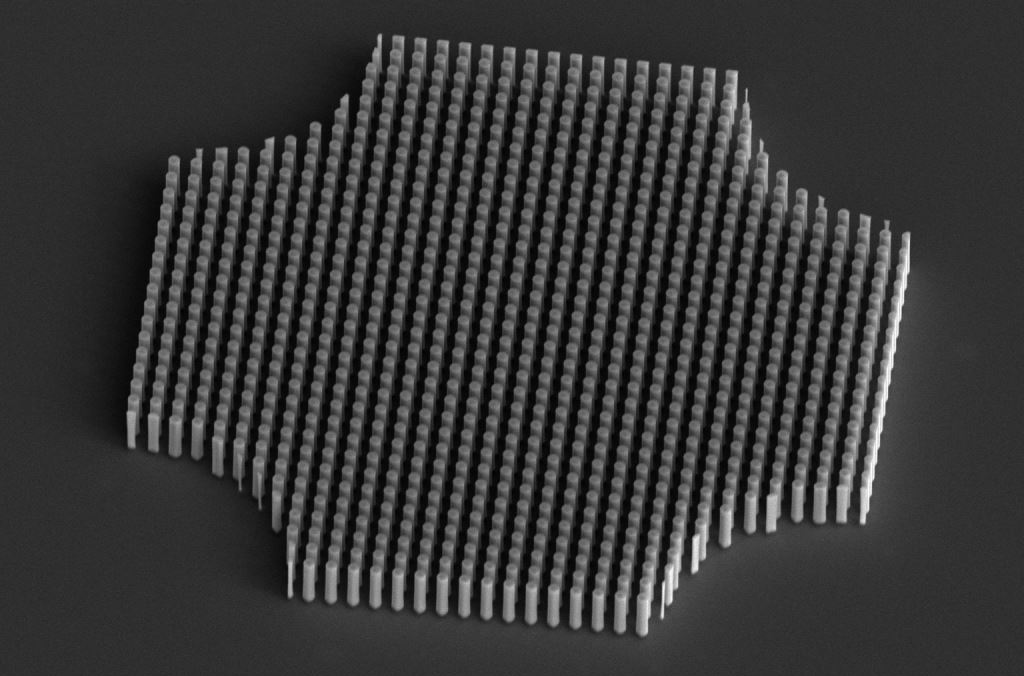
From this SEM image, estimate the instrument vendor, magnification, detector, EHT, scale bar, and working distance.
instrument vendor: FEI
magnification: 1.5 K X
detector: ETD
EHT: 10 kV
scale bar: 40000 nm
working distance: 44 mm